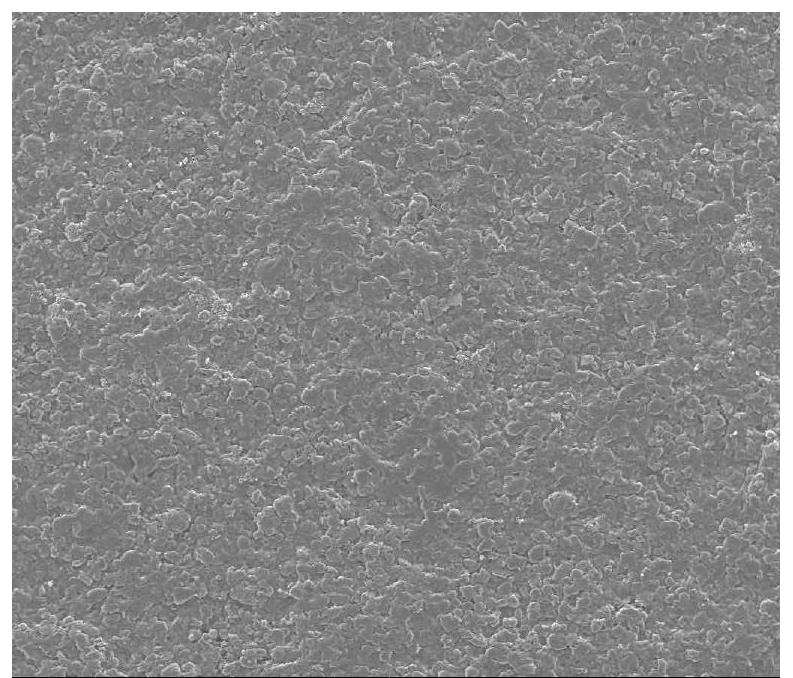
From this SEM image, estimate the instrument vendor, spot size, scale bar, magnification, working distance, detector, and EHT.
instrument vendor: FEI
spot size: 5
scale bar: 500000 nm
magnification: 0.2 K X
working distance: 11.2 mm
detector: ETD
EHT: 20 kV